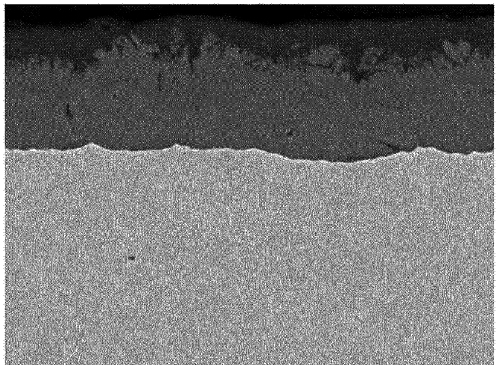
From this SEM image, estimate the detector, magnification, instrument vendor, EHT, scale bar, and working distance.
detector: COMPO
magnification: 2 K X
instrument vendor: JEOL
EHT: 20 kV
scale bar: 10000 nm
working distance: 10.1 mm